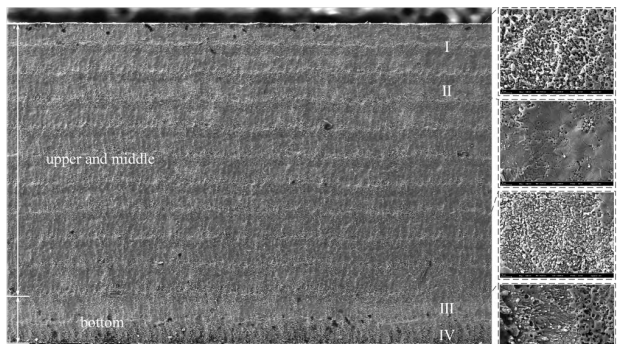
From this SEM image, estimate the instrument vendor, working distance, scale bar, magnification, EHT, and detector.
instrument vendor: Zeiss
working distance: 10.9 mm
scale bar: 200000 nm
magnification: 0.036 K X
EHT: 20 kV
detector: SE2